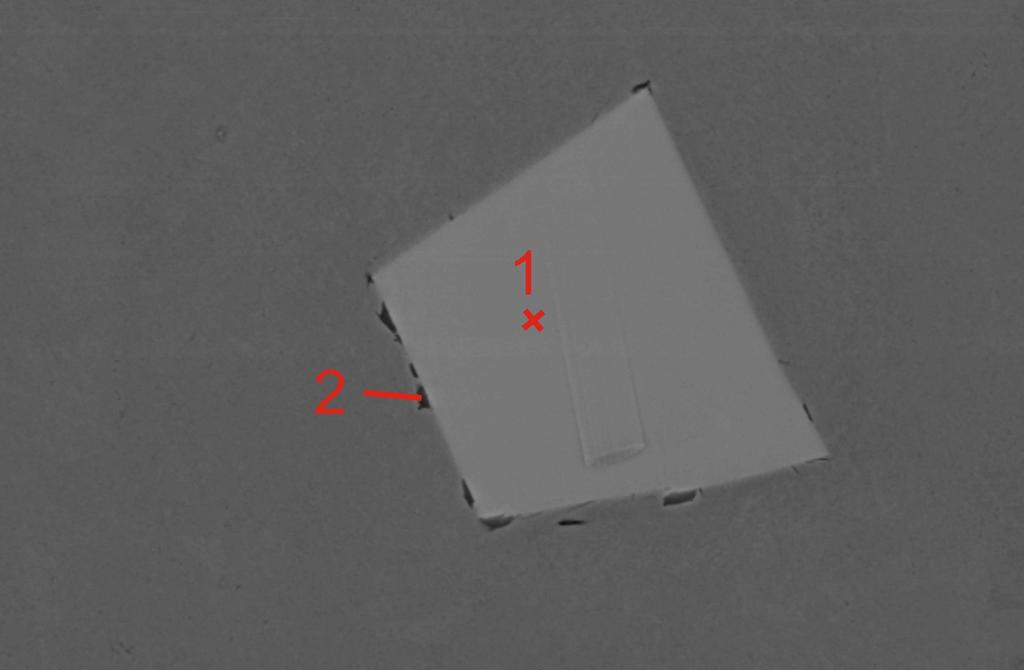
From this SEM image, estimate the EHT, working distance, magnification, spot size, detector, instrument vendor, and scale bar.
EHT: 20 kV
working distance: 11 mm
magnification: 1 K X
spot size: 4.8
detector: BSE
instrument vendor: FEI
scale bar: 20000 nm